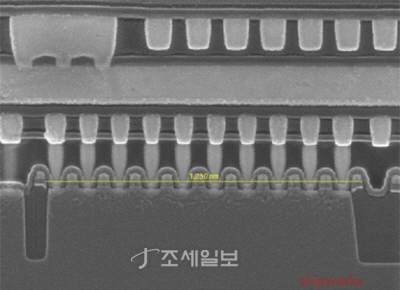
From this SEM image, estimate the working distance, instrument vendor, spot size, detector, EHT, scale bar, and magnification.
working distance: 5.1 mm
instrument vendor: FEI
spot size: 3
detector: TLD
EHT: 10 kV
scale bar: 200 nm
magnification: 80 K X